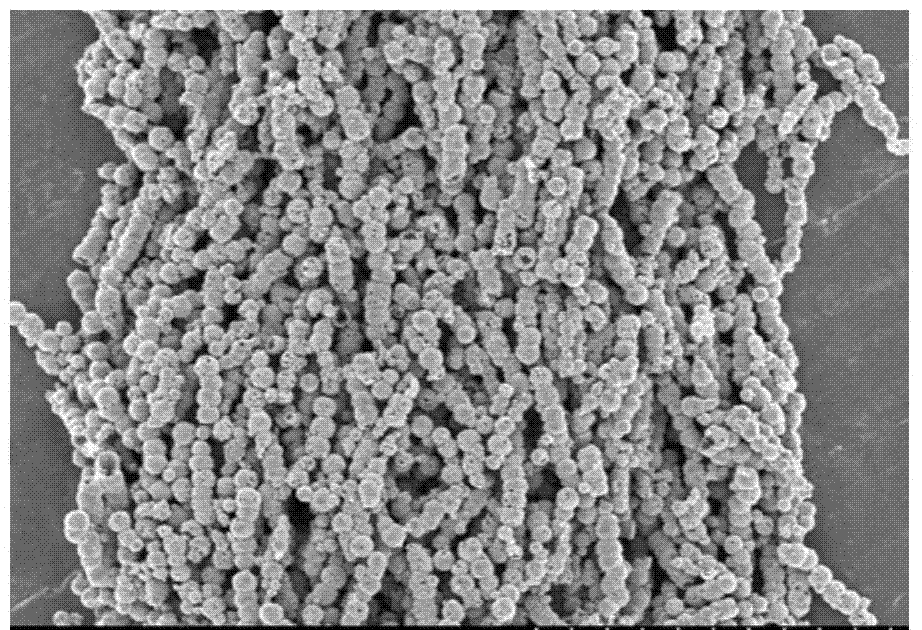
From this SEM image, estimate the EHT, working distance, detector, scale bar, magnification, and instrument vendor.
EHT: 5 kV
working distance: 6.9 mm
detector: SE(M)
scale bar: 20000 nm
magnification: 2.5 K X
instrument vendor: Hitachi